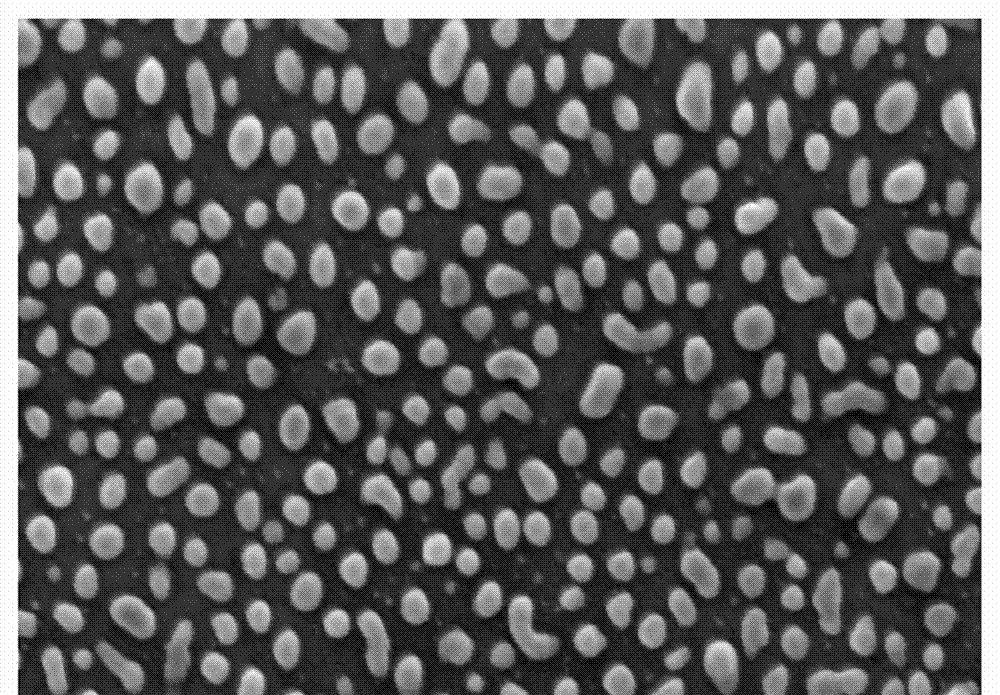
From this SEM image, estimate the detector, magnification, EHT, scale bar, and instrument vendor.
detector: SEI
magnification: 4.5 K X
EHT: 20 kV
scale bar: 5000 nm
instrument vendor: JEOL